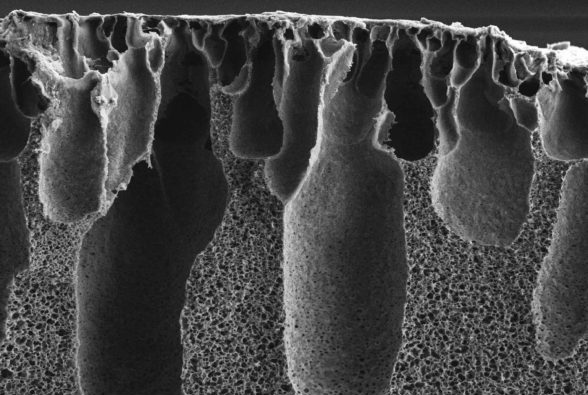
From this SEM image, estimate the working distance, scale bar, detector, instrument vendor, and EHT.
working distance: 2.9 mm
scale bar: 10000 nm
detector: SE2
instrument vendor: Zeiss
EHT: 5 kV